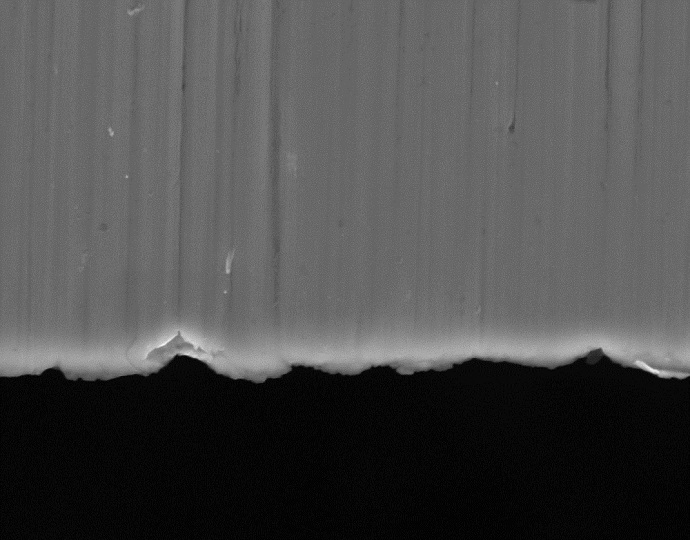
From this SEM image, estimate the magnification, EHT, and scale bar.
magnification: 5 K X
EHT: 20 kV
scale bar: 5000 nm